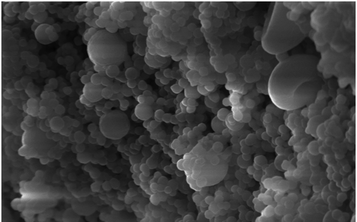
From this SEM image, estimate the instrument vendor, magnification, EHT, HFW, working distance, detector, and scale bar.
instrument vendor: Zeiss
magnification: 7.5 K X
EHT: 15 kV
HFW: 15.24 µm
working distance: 8.44 mm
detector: SE1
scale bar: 1000 nm